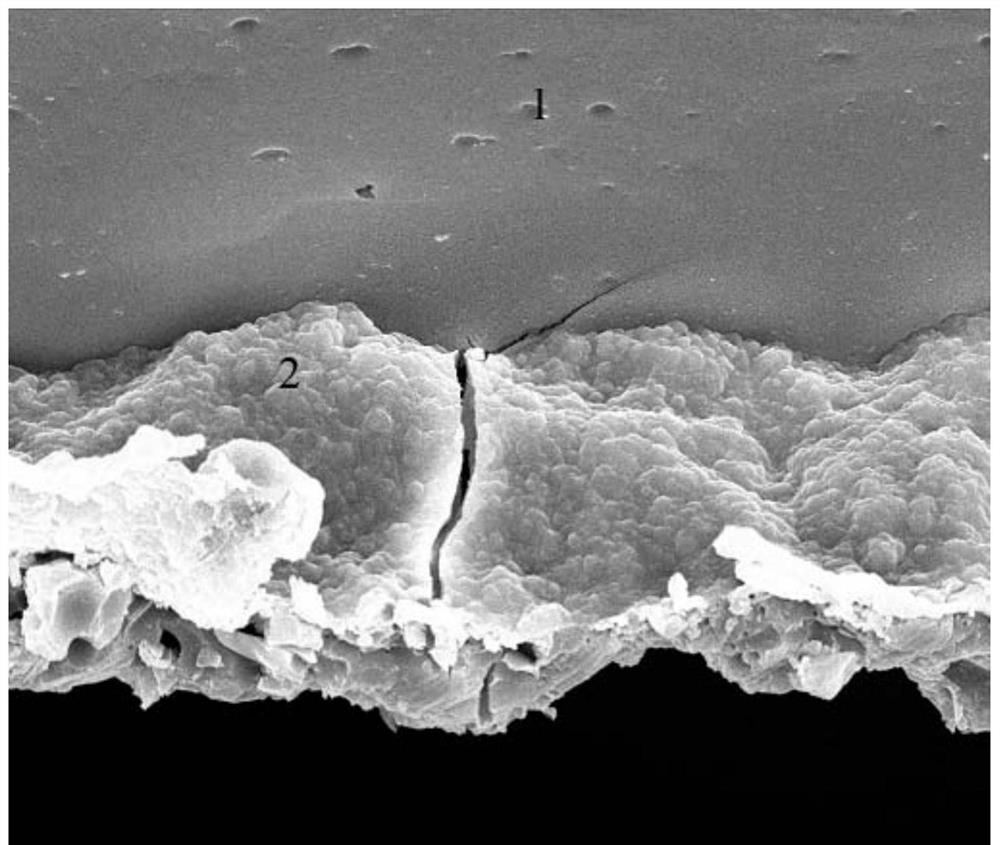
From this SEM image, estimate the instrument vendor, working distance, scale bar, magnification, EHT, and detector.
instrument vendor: FEI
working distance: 12.2 mm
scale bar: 10000 nm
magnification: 5 K X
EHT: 20 kV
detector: SE